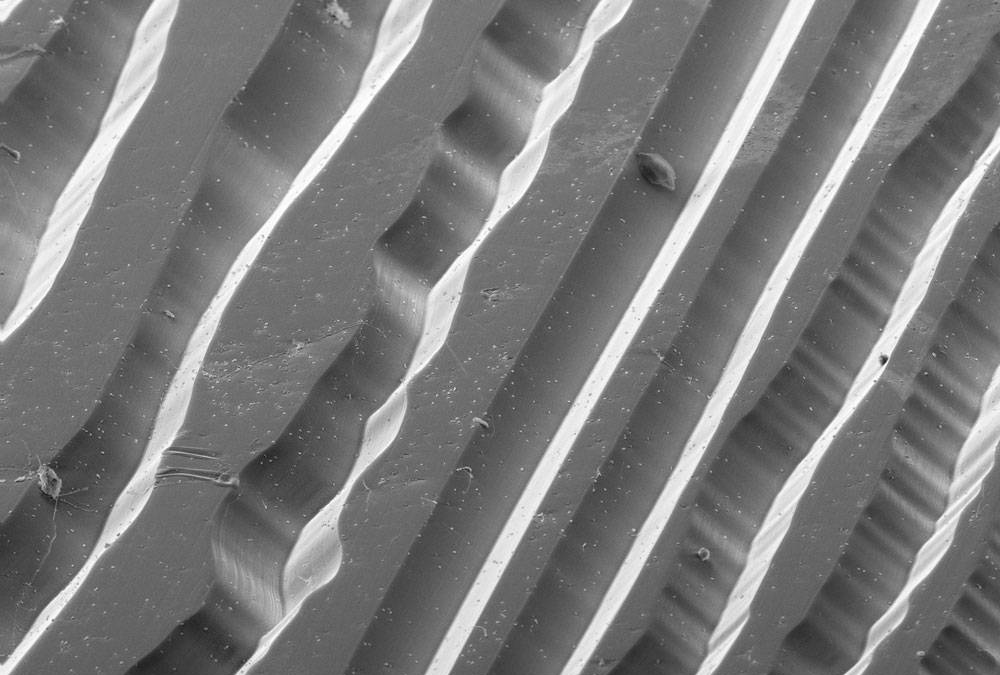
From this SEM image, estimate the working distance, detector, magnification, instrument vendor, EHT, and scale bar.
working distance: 10.5 mm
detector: SE2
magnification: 0.16 K X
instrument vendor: Zeiss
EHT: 20 kV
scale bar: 100000 nm